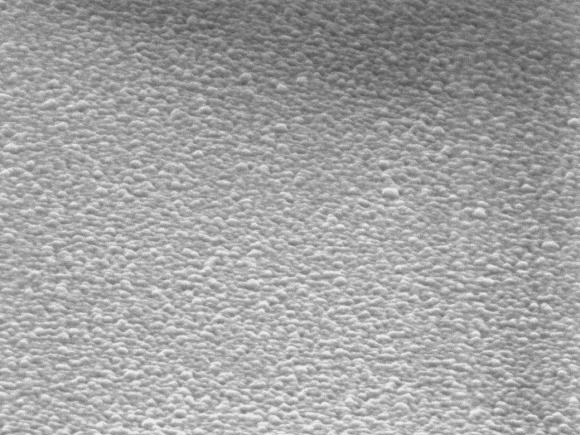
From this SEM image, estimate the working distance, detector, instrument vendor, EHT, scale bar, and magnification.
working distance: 2.3 mm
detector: SEI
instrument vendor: JEOL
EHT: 2.5 kV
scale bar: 100 nm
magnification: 100 K X